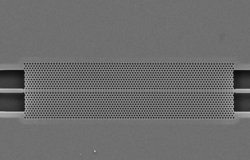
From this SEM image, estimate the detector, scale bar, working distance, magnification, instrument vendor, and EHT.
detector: SE2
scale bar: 3000 nm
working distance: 7 mm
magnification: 2.77 K X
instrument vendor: Zeiss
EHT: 5 kV